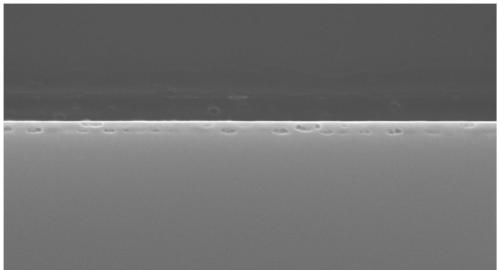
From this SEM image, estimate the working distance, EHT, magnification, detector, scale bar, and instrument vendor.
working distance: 8.6 mm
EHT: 15 kV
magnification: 50 K X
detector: SE(UL)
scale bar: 1000 nm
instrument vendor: Hitachi